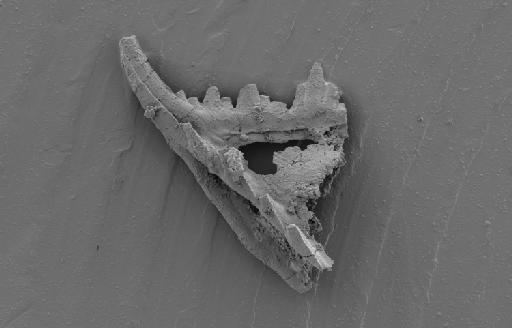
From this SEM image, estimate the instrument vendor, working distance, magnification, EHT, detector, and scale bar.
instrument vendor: Zeiss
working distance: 7.2 mm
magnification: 0.33 K X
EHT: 3 kV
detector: SE2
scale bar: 100000 nm